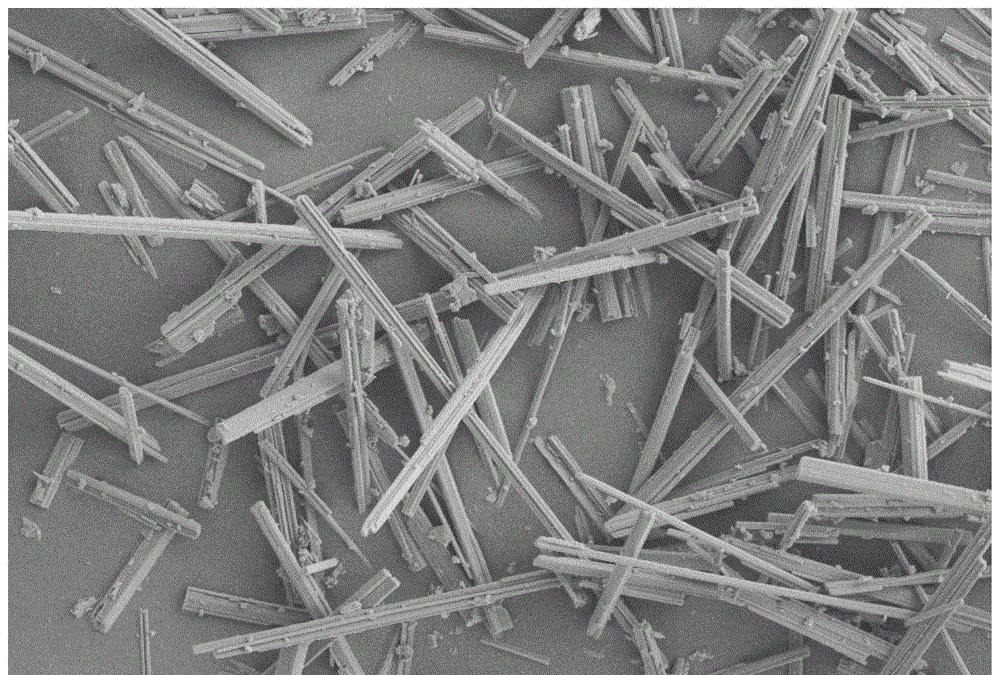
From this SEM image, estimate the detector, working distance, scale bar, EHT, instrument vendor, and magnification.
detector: SE2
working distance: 7 mm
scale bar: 10000 nm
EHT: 5 kV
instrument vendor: Zeiss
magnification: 1 K X